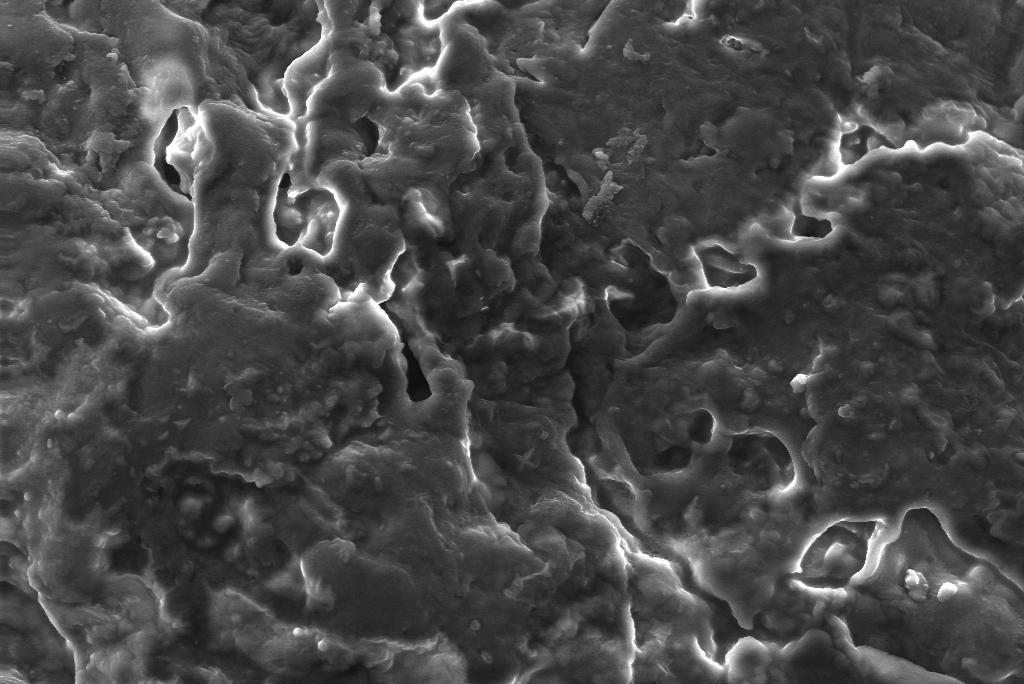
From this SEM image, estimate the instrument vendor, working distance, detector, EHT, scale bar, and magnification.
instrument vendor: Zeiss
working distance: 10 mm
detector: SE1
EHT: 20 kV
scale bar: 10000 nm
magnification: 1 K X